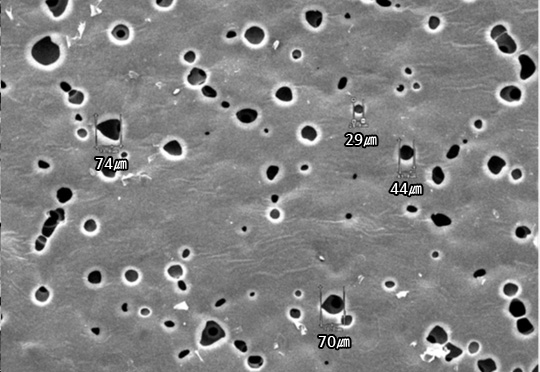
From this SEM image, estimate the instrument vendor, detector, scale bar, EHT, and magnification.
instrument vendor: other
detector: SED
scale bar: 500000 nm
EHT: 30 kV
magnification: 0.08 K X